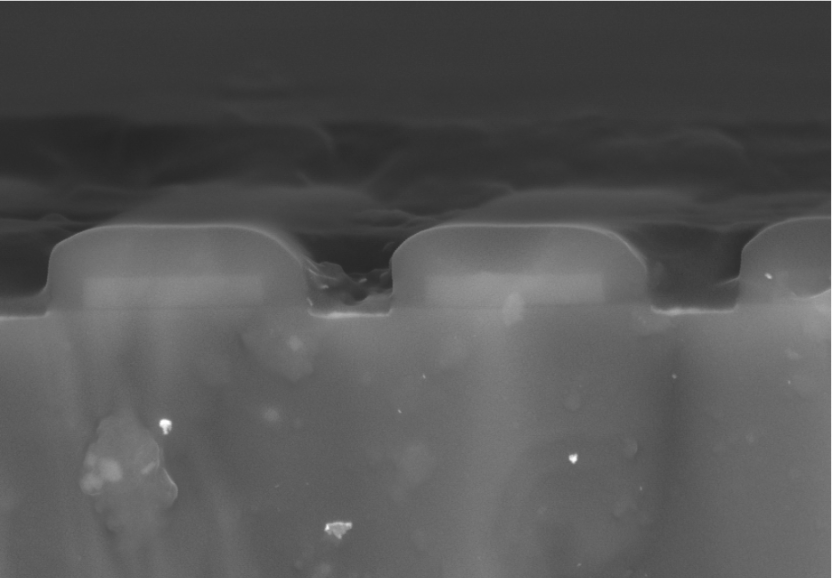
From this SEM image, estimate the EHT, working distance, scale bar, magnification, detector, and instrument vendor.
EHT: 20 kV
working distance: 6.565 mm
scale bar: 3000 nm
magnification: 10 K X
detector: SE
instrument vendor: other